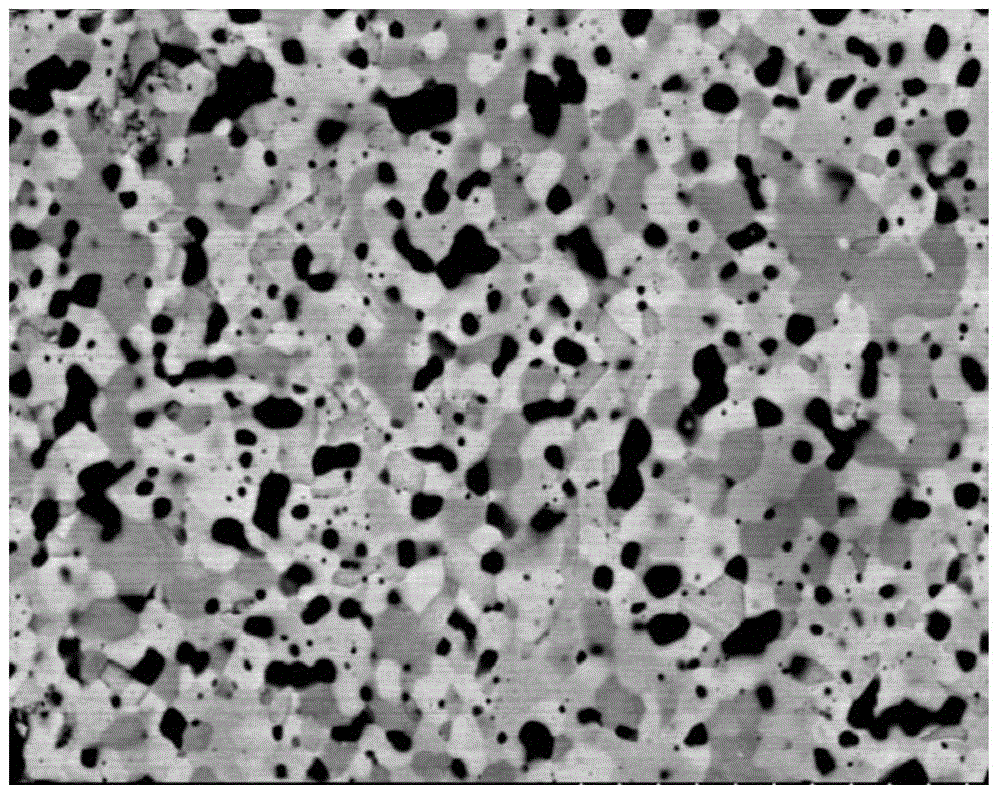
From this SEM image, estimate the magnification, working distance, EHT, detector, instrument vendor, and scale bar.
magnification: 5 K X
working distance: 11.9 mm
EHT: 15 kV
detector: YAGBSE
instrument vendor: Hitachi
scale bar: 10000 nm